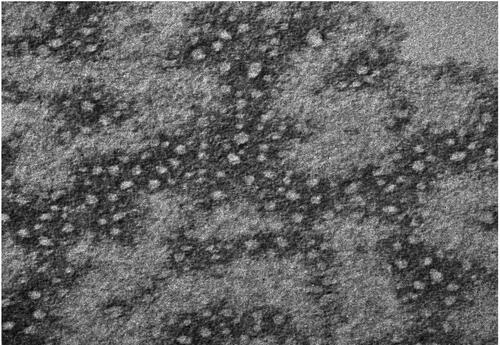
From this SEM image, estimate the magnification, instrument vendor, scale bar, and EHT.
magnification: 100 K X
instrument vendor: other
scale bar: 100 nm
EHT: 80 kV